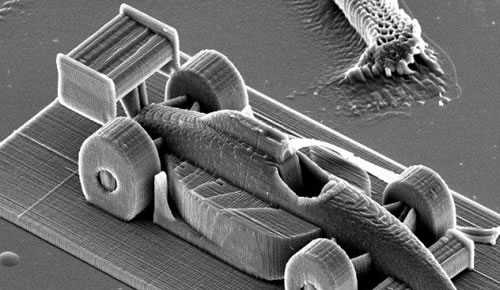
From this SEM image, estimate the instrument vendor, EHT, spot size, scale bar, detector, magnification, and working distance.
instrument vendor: FEI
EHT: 20 kV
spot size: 4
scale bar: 100000 nm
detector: SE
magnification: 0.364 K X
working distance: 27.2 mm